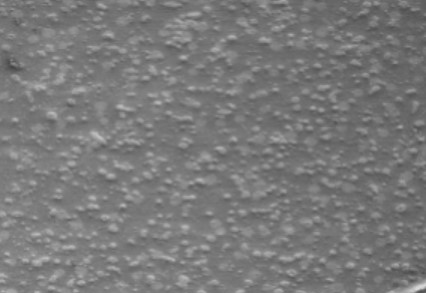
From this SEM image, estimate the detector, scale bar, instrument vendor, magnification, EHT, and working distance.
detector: SE(M)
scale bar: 1000000 nm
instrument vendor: Hitachi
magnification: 0.05 K X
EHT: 2 kV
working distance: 13 mm